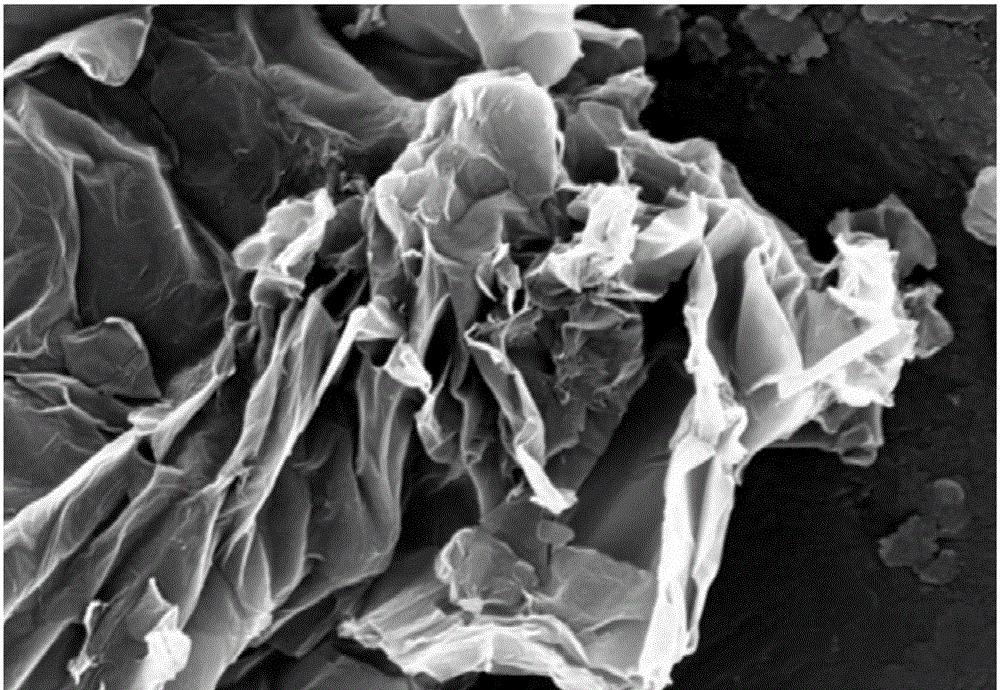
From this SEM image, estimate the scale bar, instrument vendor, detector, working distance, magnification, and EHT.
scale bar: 200 nm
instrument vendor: Zeiss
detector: InLens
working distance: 4.4 mm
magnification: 72.5 K X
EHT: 5 kV